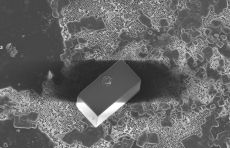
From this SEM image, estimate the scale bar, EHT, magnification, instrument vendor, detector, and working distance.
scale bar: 10000 nm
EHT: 5 kV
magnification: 5.33 K X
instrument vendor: Zeiss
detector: InLens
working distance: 6 mm